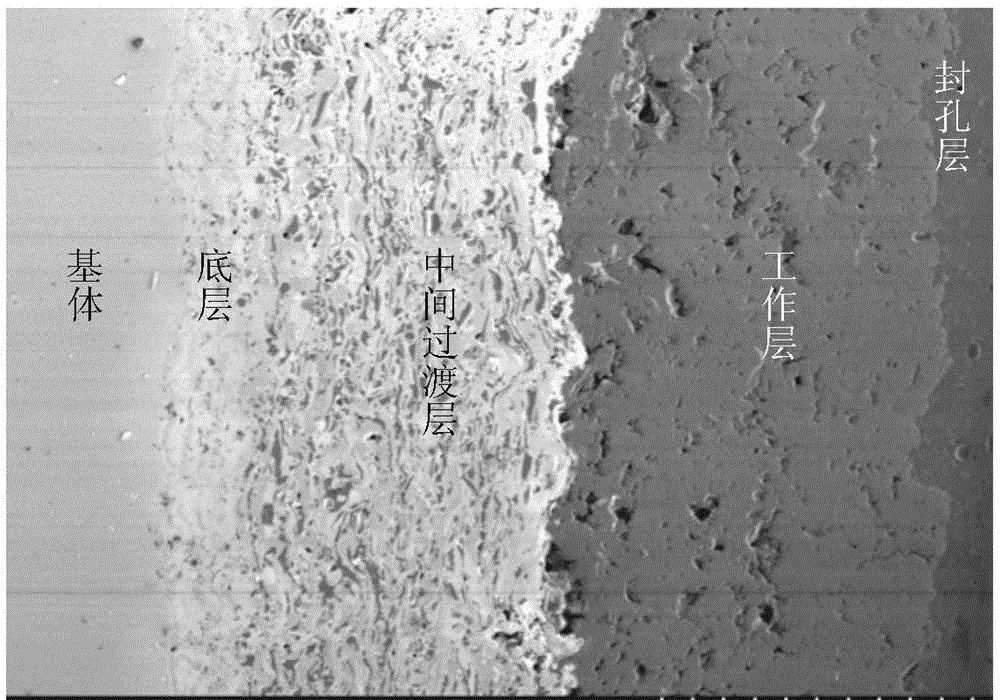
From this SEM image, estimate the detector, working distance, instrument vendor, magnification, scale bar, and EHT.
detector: SE(M)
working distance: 15.4 mm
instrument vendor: Hitachi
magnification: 0.2 K X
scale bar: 200000 nm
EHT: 1 kV